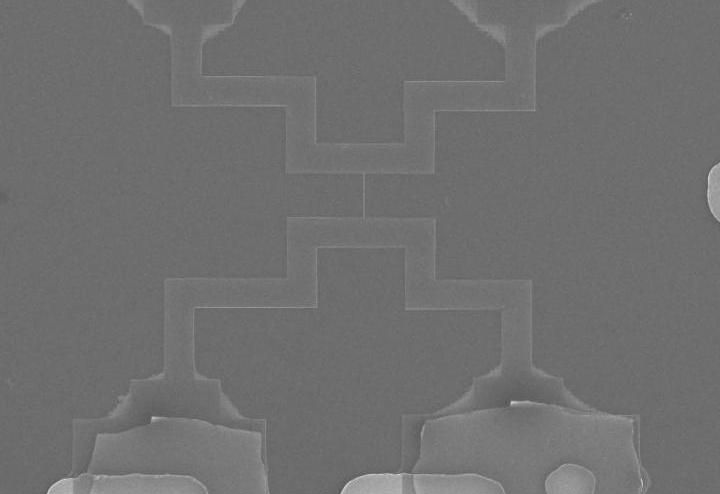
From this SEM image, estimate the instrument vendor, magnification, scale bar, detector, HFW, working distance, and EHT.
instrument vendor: FEI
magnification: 6.195 K X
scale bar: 10000 nm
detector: ETD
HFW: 49.5 µm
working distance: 5.2 mm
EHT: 10 kV